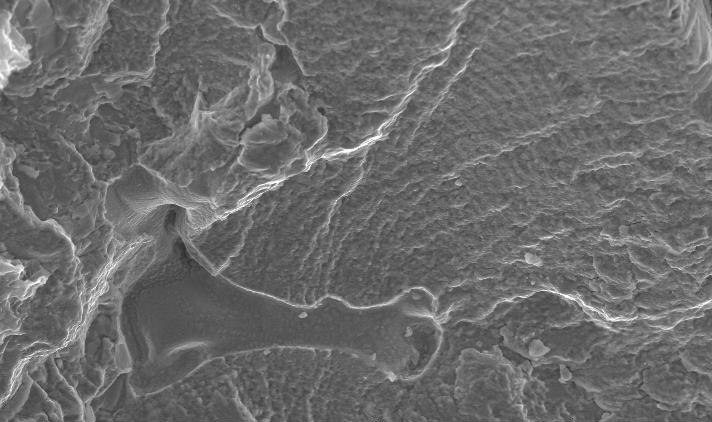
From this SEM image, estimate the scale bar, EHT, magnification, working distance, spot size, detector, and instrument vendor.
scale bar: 50000 nm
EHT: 20 kV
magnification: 0.5 K X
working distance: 9.7 mm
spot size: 4.3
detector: SE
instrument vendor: FEI